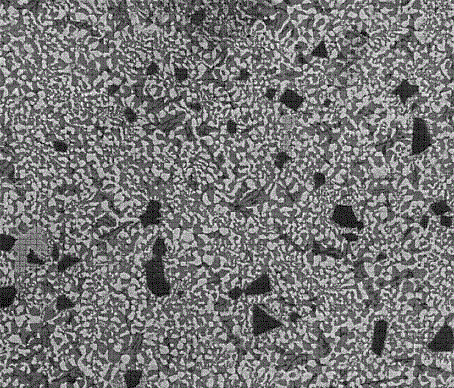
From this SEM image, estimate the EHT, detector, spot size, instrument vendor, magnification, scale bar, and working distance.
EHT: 20 kV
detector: ETD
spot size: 2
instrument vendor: FEI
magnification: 1 K X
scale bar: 100000 nm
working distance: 12.4 mm